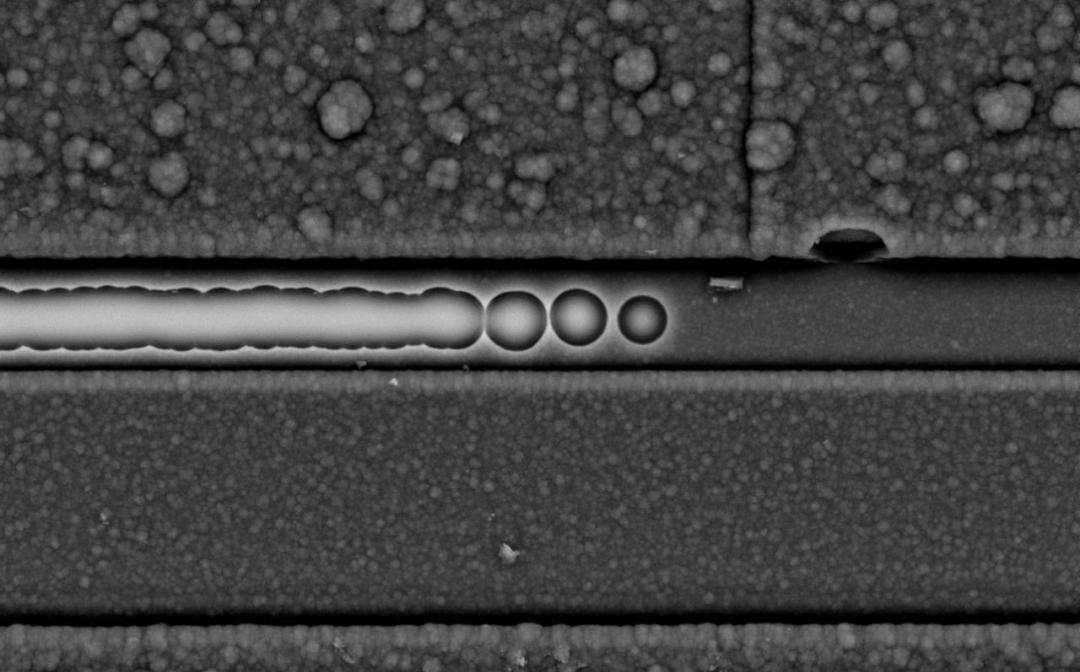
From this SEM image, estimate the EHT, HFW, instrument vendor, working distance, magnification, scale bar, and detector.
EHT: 10 kV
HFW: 42.3 µm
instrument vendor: other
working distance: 9.552 mm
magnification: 3 K X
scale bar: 10000 nm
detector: BSD Full